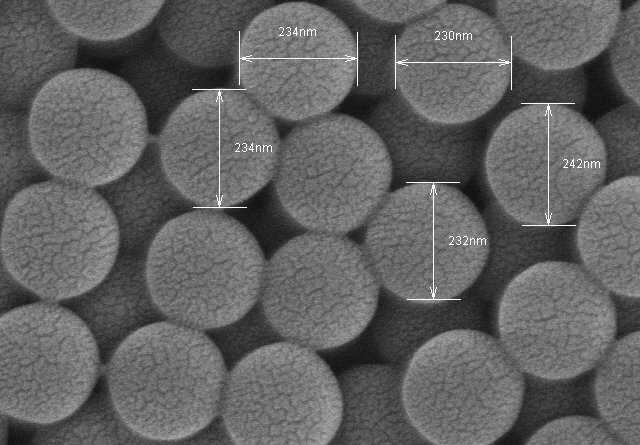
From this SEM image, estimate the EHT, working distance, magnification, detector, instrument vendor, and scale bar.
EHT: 7 kV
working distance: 8.5 mm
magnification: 100 K X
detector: SE(M)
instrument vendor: Hitachi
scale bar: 500 nm